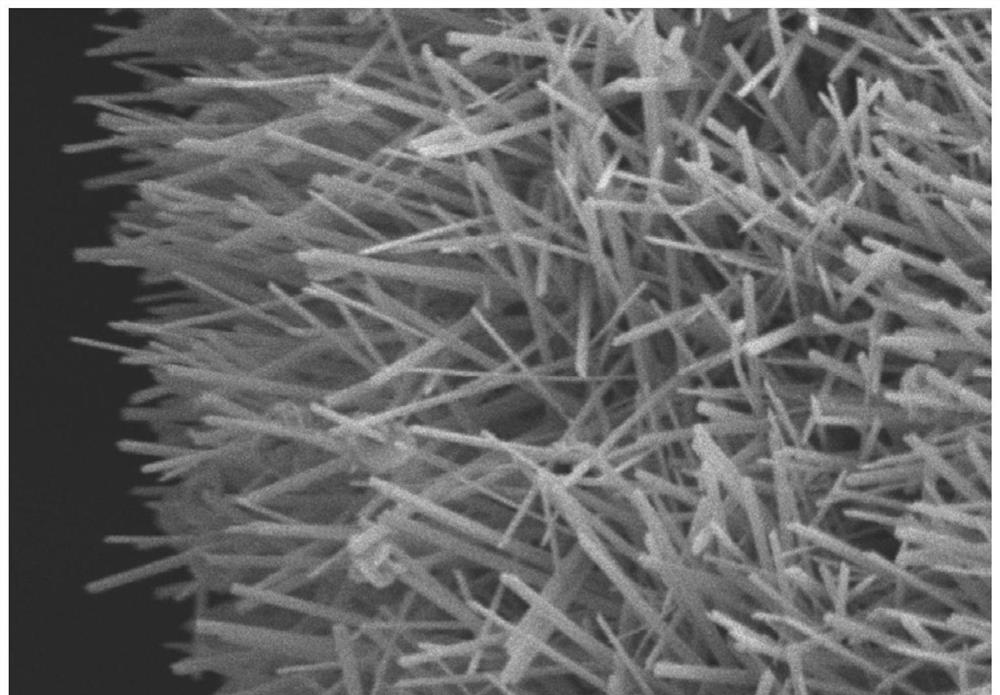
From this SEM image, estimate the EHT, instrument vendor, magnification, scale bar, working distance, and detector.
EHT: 5 kV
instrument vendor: Hitachi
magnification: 8 K X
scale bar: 5000 nm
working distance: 11.8 mm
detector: SE